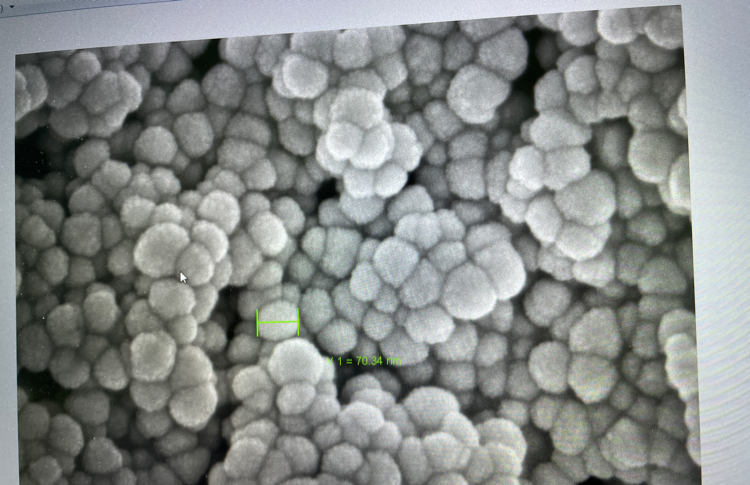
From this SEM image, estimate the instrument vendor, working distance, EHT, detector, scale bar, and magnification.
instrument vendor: Zeiss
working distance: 6.4 mm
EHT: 5 kV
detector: InLens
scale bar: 100 nm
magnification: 100 K X